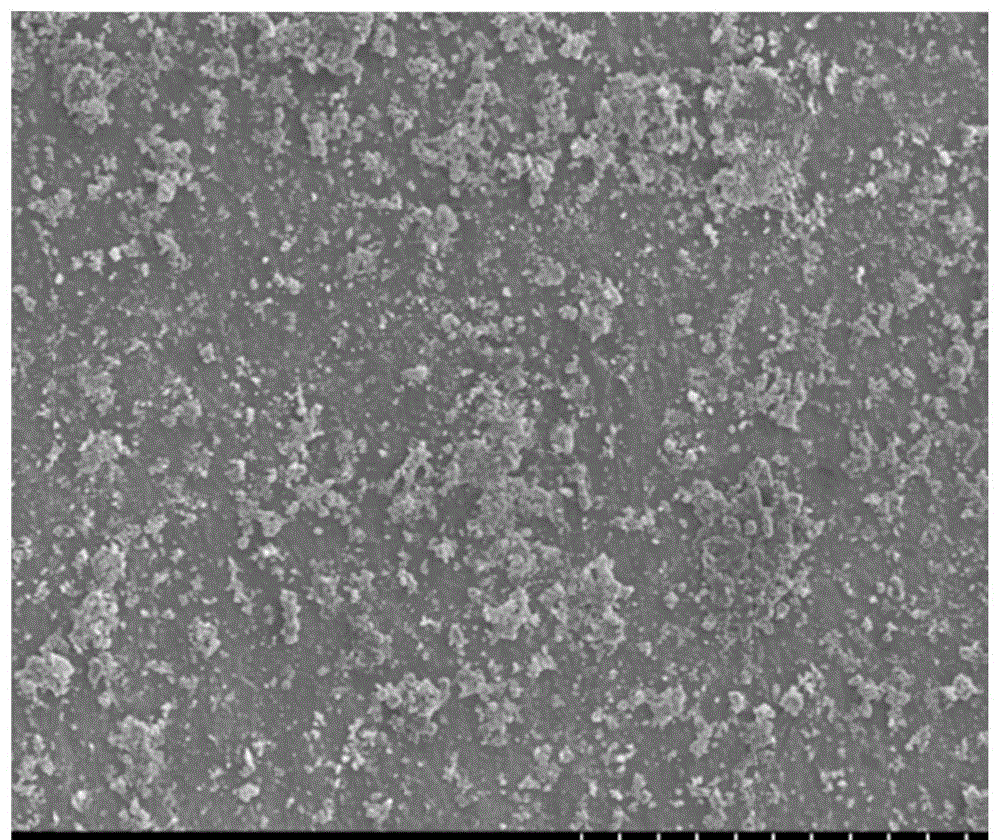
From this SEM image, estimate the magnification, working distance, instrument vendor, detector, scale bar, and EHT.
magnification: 1 K X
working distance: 8.9 mm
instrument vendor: Hitachi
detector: SE(M)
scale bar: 50000 nm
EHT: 5 kV